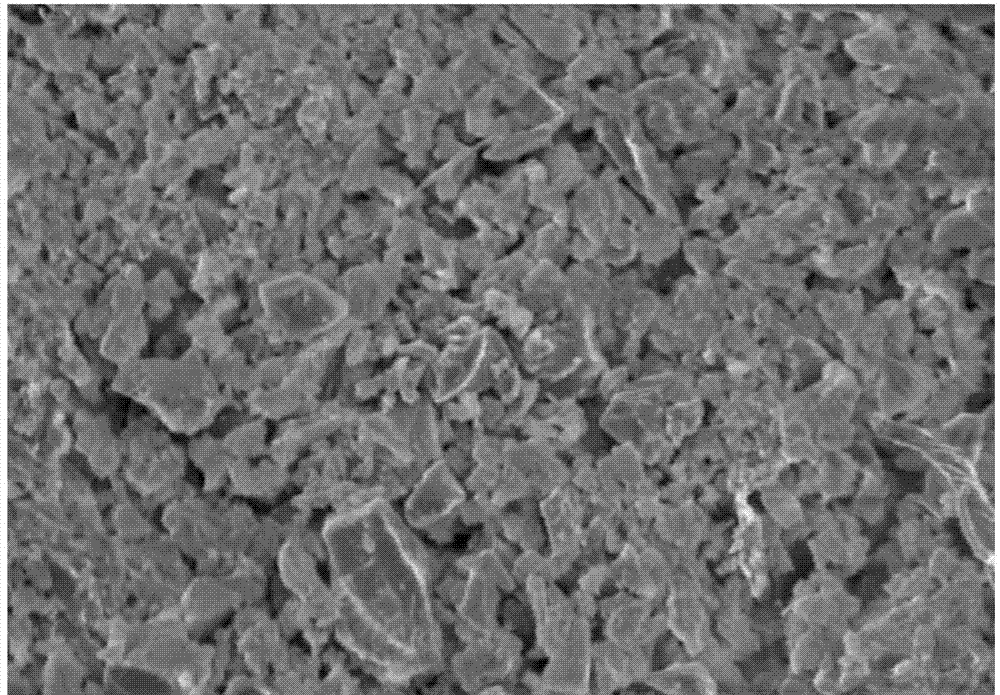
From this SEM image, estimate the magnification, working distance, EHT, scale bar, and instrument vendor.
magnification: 5 K X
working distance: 14.1 mm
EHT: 5 kV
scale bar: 10000 nm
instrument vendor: Hitachi